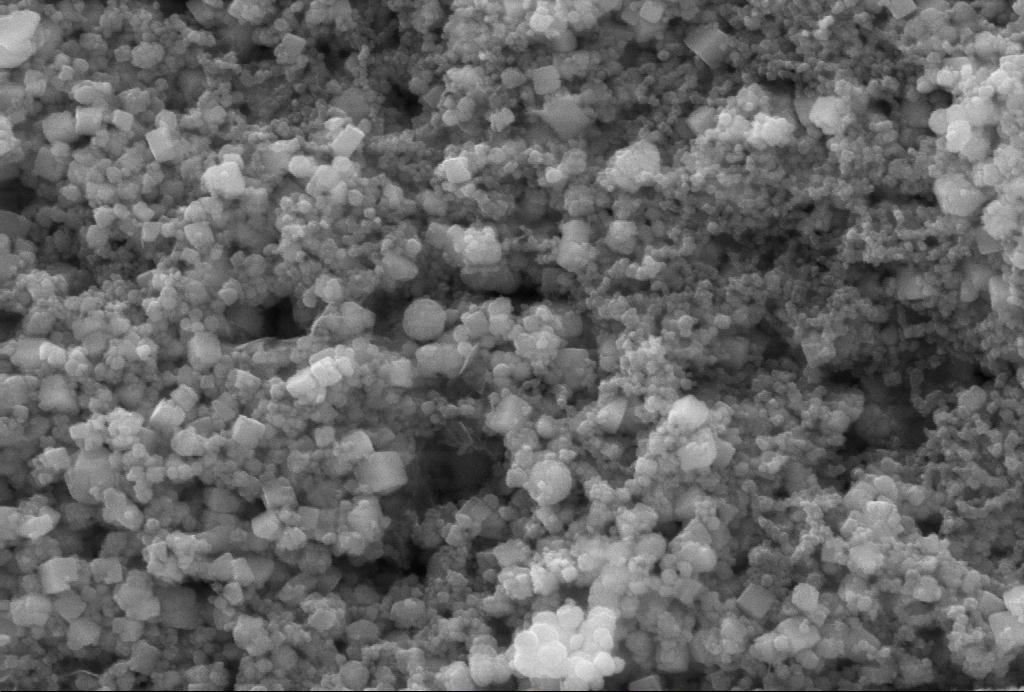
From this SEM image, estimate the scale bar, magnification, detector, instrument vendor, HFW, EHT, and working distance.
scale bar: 100 nm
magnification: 100 K X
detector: SE2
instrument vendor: Zeiss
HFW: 3.811 µm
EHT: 10 kV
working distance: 9.4 mm